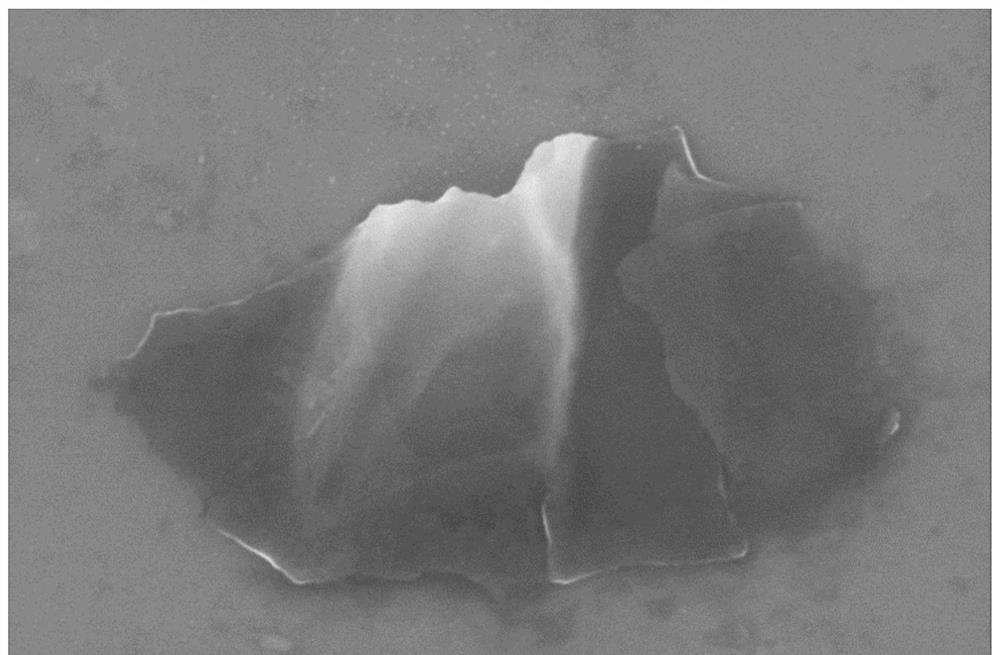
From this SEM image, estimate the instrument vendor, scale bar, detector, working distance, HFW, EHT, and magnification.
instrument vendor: FEI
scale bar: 1000 nm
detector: TLD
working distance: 2.9 mm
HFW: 4.14 µm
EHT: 5 kV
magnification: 50 K X